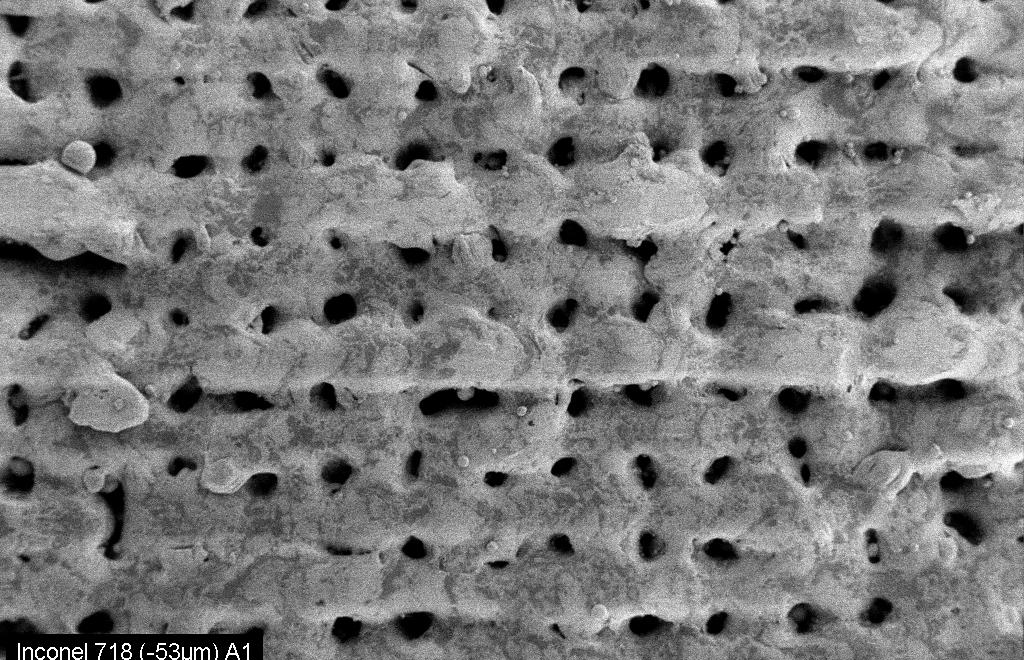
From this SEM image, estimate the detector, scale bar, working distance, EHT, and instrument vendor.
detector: SE2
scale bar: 100000 nm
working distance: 10 mm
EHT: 10 kV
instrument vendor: Zeiss